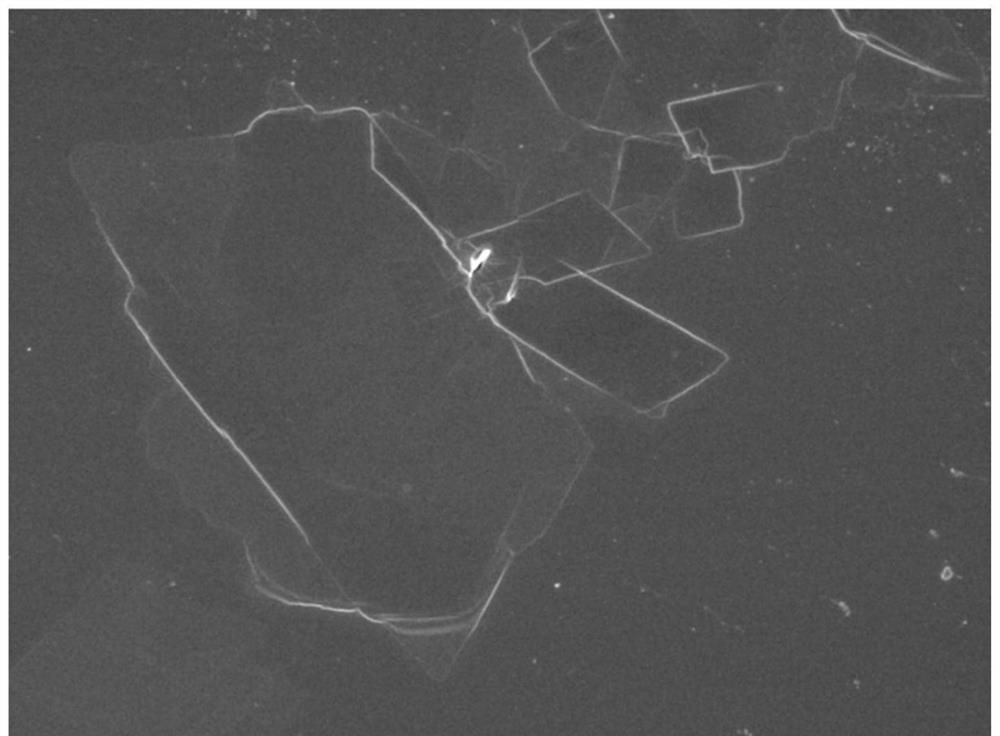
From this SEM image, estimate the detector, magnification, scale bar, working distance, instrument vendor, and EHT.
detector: SEI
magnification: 2.7 K X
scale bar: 10000 nm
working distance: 8.4 mm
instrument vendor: JEOL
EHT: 5 kV